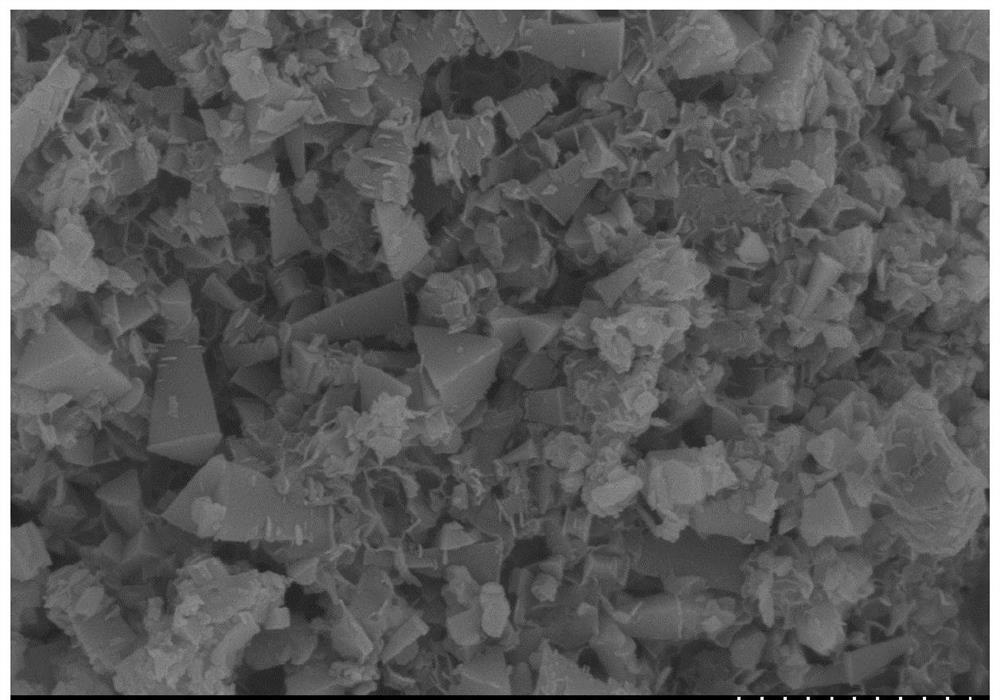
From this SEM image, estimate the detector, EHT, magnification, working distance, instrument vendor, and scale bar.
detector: SE(M)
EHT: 5 kV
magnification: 30 K X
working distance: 10.8 mm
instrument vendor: Hitachi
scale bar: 1000 nm